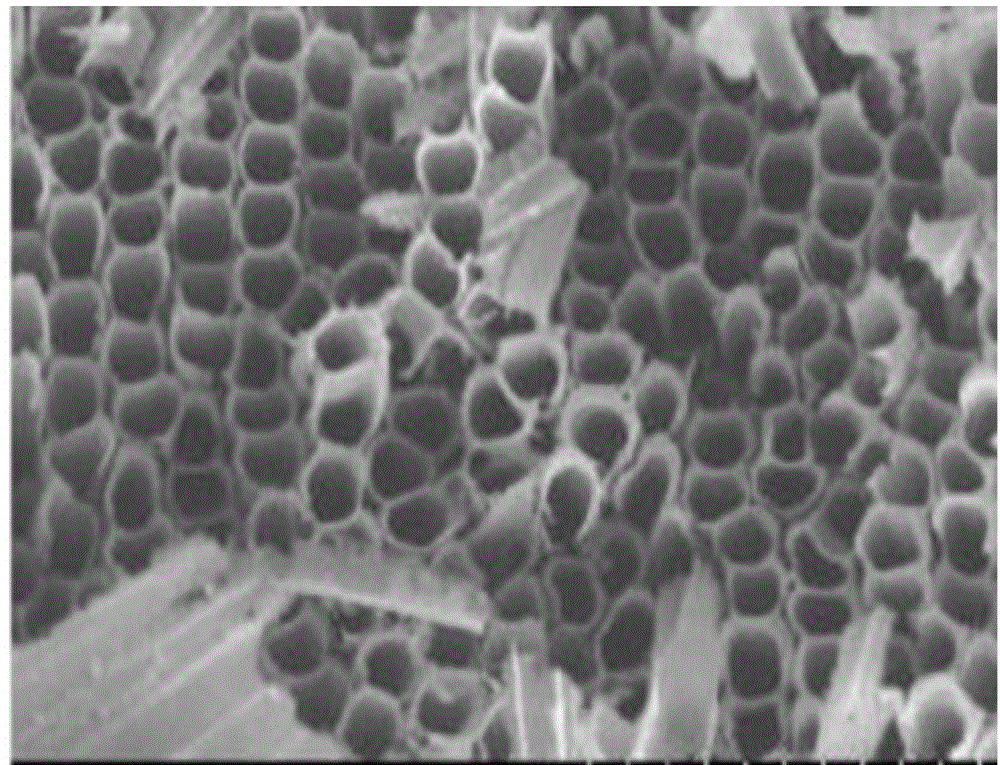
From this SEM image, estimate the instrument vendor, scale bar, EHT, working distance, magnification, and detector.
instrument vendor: Hitachi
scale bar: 1000 nm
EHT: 3 kV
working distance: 5.1 mm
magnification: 50 K X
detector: SE(U)